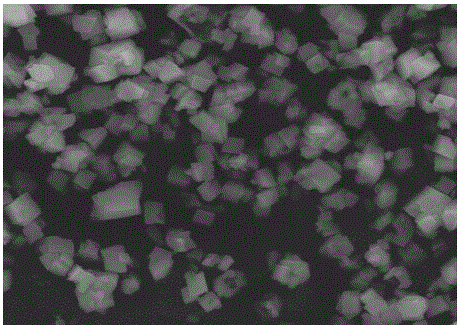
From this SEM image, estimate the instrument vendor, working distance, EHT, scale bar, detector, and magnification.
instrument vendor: JEOL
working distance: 10 mm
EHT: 20 kV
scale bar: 1000 nm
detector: LED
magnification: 3.5 K X